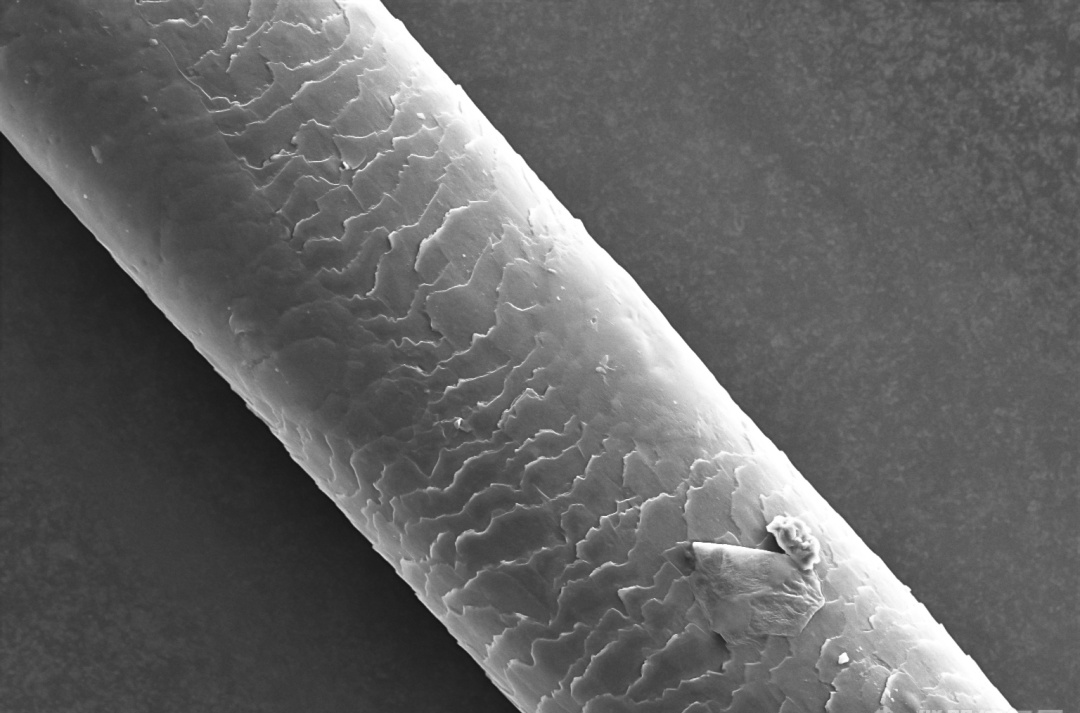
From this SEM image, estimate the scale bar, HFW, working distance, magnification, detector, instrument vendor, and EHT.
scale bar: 50000 nm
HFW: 254 µm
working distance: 8.1 mm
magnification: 0.5 K X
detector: ETD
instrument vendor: Thermo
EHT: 15 kV